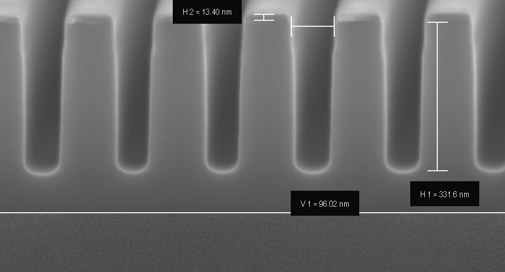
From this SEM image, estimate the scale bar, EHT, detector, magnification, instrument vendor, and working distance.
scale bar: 200 nm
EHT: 5 kV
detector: InLens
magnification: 100 K X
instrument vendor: Zeiss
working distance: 3 mm